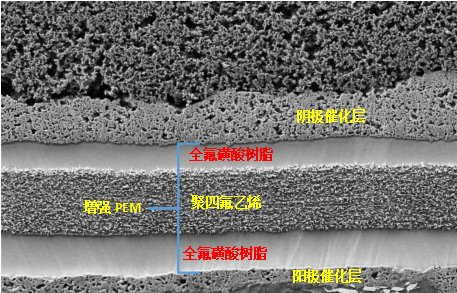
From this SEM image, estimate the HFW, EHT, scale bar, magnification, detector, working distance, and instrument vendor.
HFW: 25.4 µm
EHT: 2 kV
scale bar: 10000 nm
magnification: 5 K X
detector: ETD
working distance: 7.4 mm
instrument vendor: Thermo